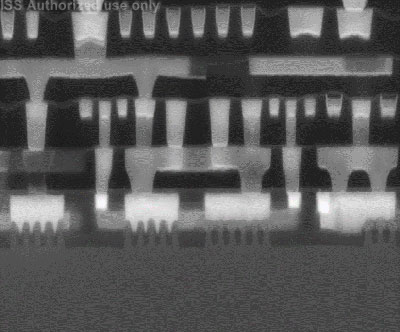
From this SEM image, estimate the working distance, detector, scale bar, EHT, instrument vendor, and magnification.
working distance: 4.9 mm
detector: vCD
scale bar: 500 nm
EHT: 10 kV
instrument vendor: FEI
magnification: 120 K X